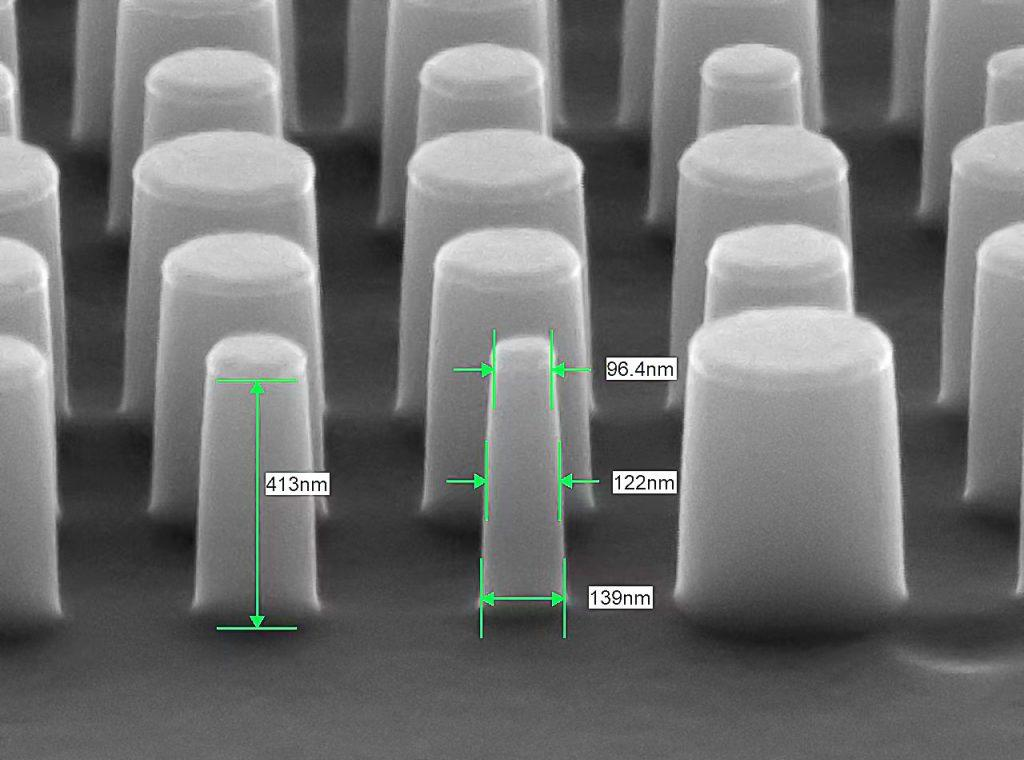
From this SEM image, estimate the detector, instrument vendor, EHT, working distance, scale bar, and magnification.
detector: LED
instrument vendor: JEOL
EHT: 10 kV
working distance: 8.8 mm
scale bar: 100 nm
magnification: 70 K X